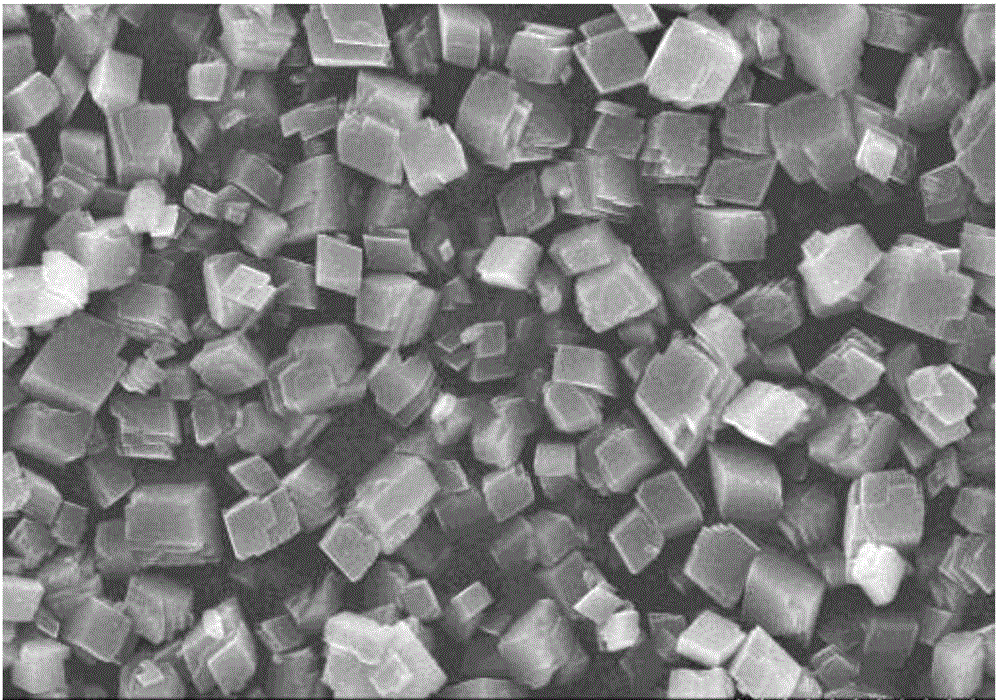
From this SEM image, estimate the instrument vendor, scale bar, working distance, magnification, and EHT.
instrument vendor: Hitachi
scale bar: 10000 nm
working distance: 12.5 mm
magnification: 3 K X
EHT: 20 kV